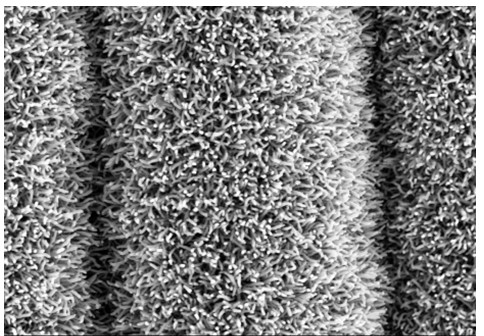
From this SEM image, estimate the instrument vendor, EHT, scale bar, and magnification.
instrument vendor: Hitachi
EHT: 5 kV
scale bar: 5000 nm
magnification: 10 K X